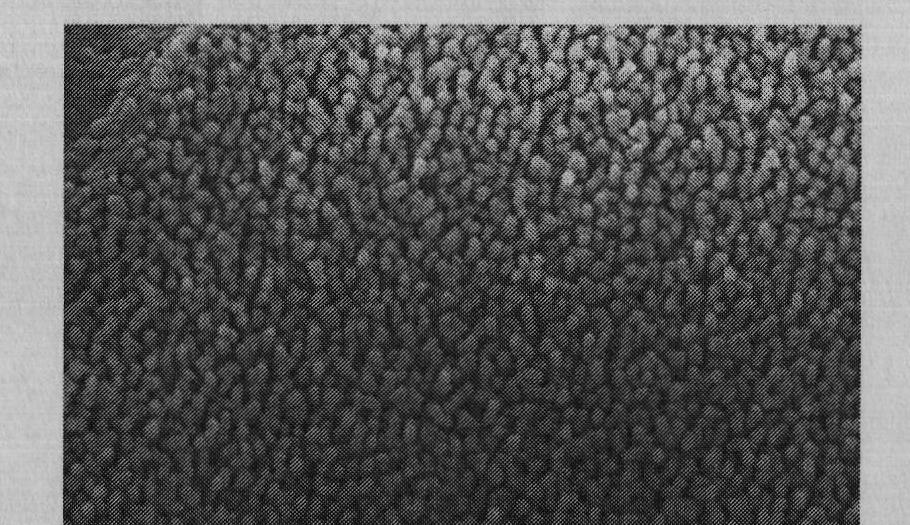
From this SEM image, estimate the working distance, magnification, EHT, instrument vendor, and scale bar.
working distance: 8 mm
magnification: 50 K X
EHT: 20 kV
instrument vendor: Zeiss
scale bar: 1000 nm